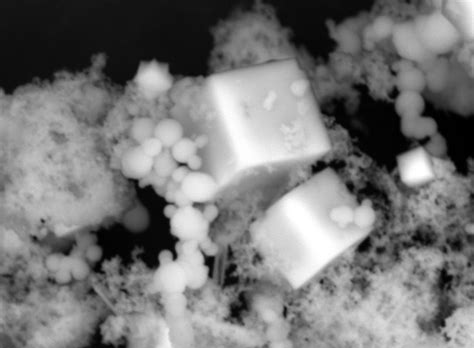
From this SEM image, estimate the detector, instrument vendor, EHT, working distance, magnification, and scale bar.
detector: BED-C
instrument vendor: JEOL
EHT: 20 kV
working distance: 10 mm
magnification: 6 K X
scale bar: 2000 nm